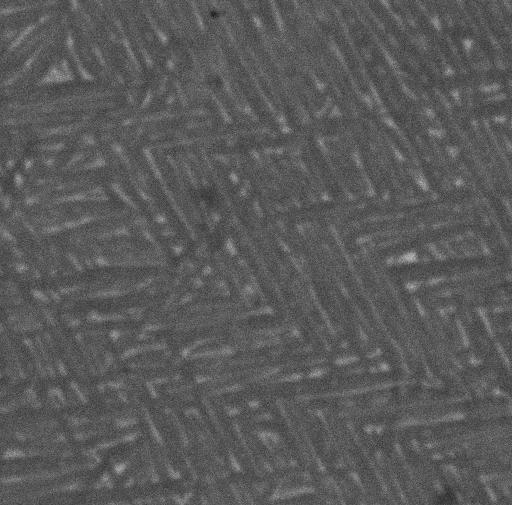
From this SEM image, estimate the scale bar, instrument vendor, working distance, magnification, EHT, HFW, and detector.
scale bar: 5000 nm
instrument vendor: Tescan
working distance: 21.15 mm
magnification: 4.5 K X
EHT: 20 kV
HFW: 28.2 µm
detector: BSE